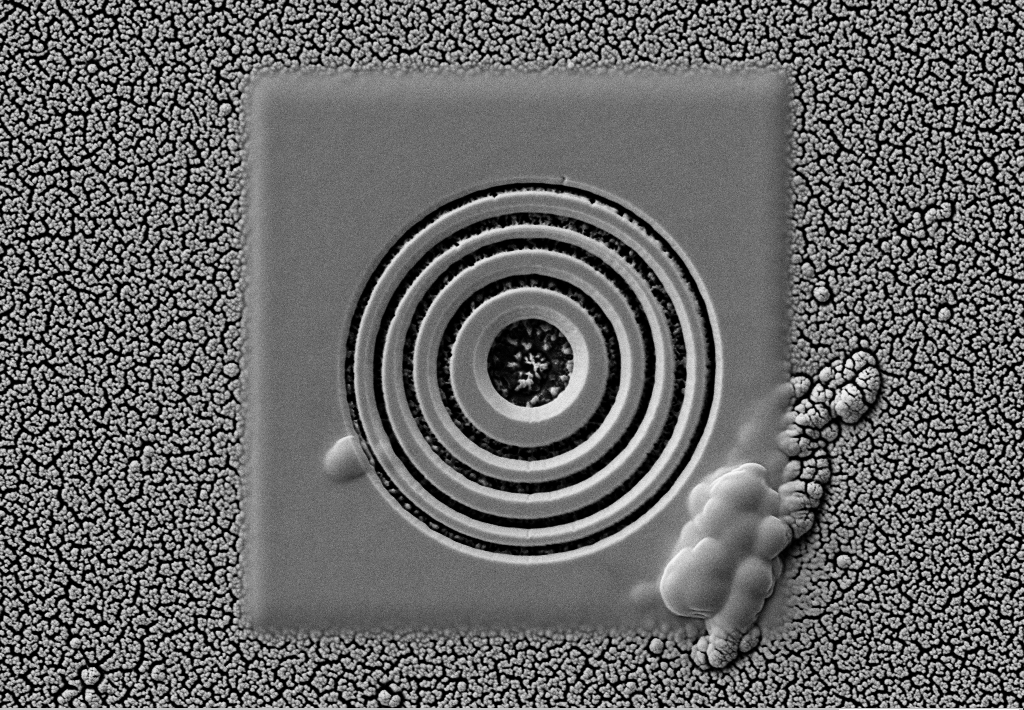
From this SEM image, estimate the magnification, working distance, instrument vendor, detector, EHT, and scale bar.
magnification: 5 K X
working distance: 8.3 mm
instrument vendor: Zeiss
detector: SE2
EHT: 5 kV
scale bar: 1000 nm